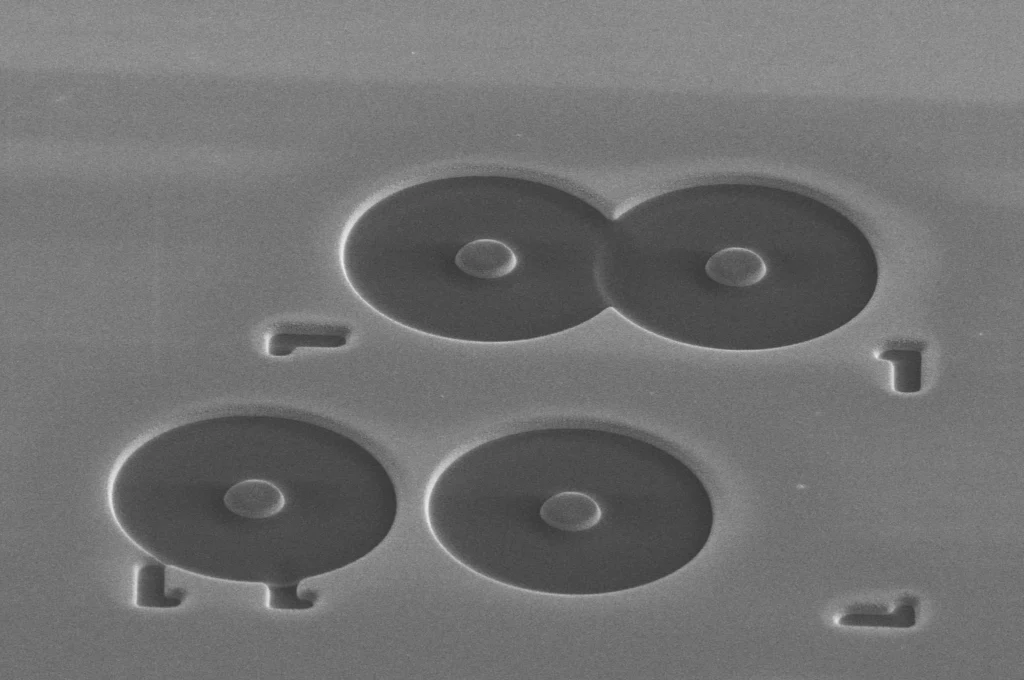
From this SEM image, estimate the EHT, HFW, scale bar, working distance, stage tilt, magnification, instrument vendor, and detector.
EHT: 2 kV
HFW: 59.2 µm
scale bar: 10000 nm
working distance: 3.9 mm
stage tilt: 52°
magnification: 3.5 K X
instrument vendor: FEI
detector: ETD